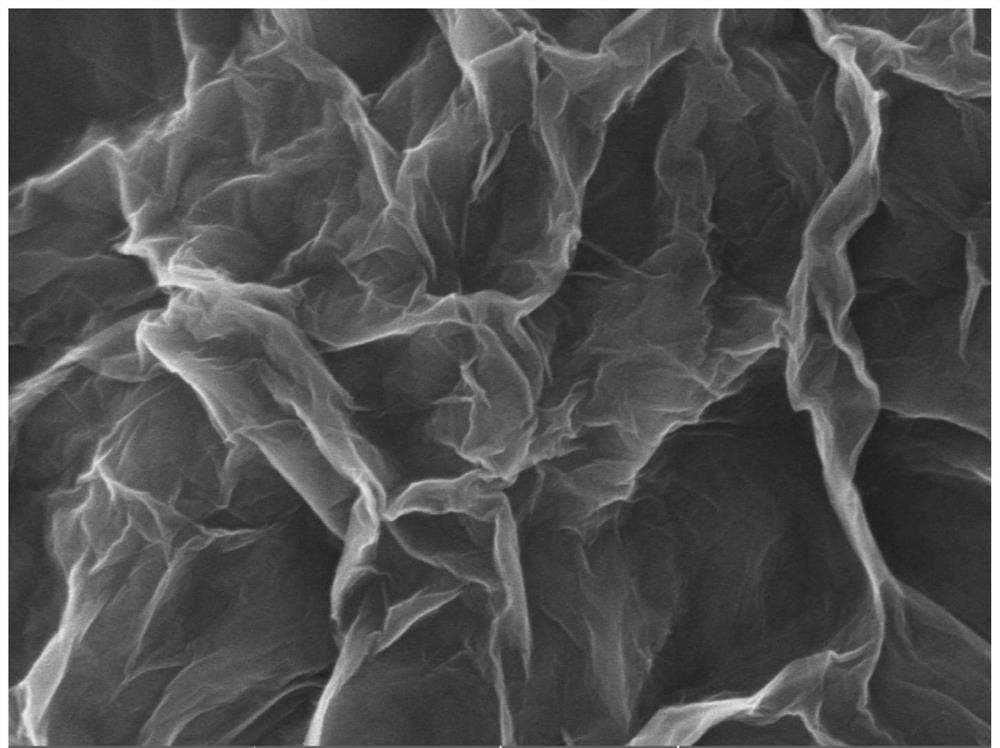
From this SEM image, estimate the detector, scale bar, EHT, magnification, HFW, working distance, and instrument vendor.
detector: In-Beam SE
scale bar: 500 nm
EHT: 5 kV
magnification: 100 K X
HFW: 2.77 µm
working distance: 3.98 mm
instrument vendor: Tescan